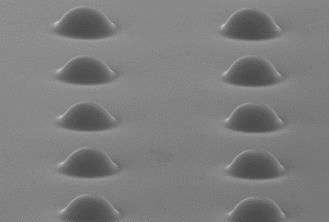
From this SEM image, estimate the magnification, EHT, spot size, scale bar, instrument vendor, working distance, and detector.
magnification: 0.6 K X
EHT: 10 kV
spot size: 3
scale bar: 50000 nm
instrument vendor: FEI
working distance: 15.4 mm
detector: SE